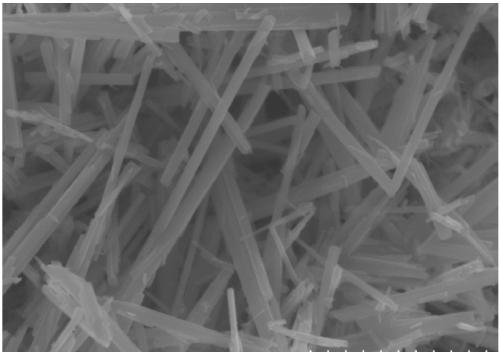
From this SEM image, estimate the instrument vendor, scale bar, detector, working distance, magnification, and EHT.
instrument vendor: Hitachi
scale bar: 1000 nm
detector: SE(UL)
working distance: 9.6 mm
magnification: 45 K X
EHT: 5 kV